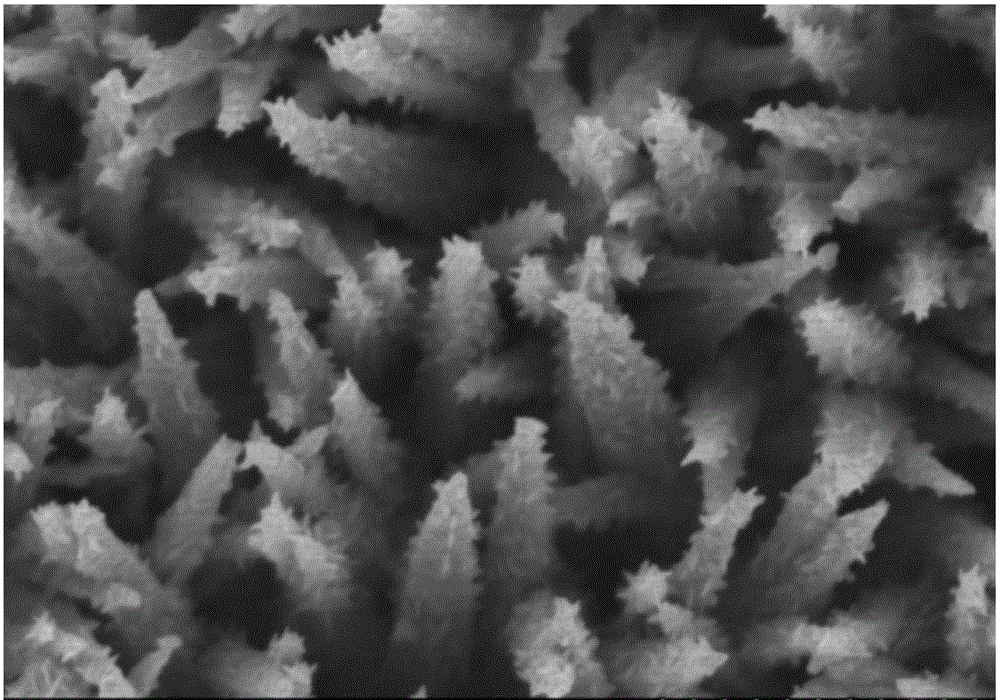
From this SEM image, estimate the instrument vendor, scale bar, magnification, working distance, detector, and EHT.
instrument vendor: Hitachi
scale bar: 1000 nm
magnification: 40 K X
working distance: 8.5 mm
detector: SE(M)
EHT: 3 kV